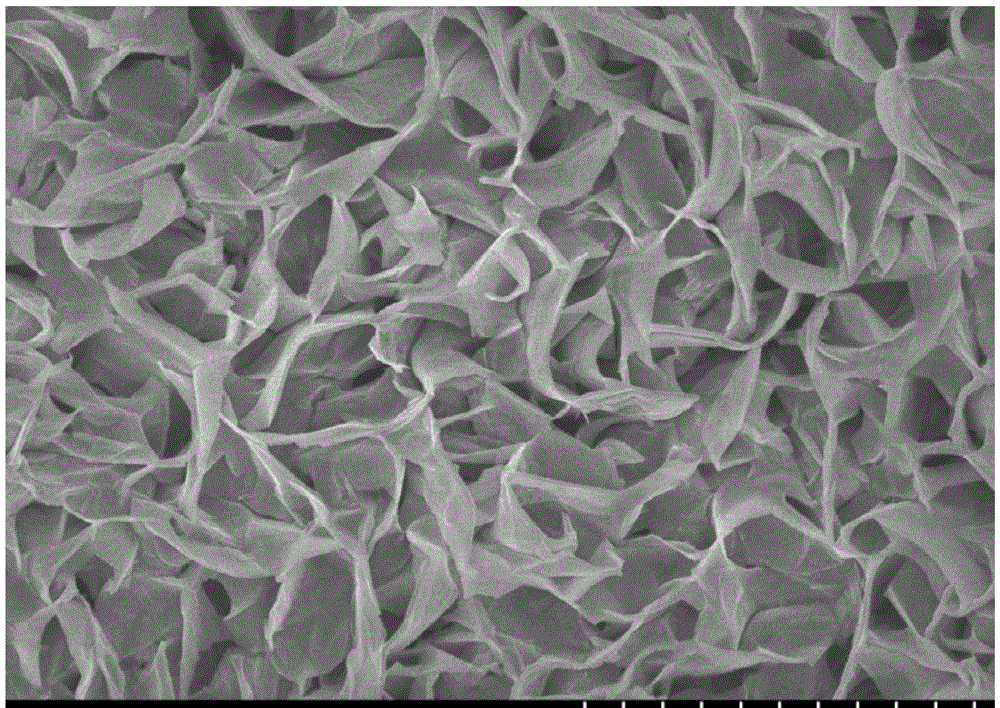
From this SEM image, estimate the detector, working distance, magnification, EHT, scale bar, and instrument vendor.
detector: SE(U)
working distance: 10.2 mm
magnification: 10 K X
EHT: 4 kV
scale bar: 5000 nm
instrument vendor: Hitachi